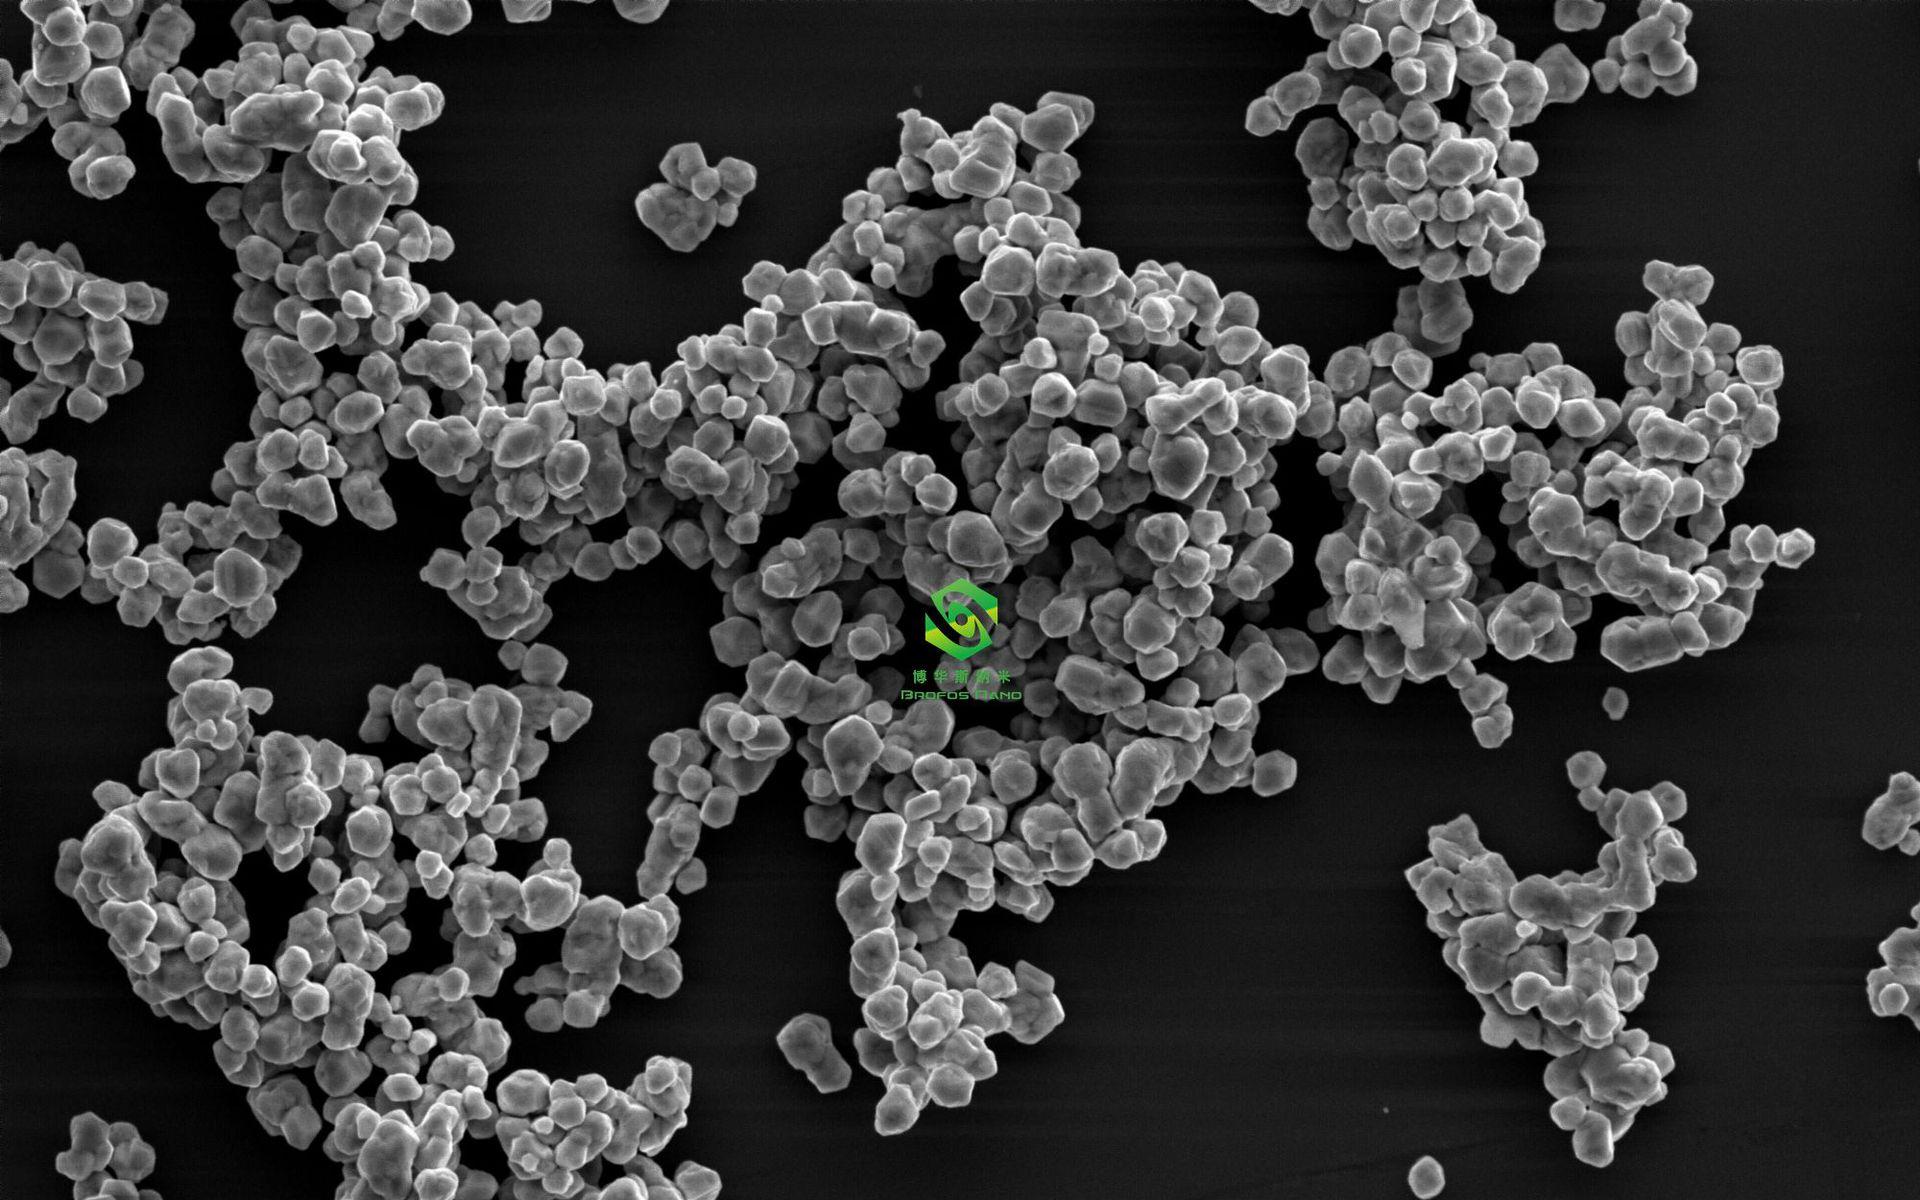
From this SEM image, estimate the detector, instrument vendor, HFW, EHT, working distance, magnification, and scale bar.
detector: SED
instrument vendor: Phenom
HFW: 39.3 µm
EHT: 10 kV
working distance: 7.529 mm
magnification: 13 K X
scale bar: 10000 nm